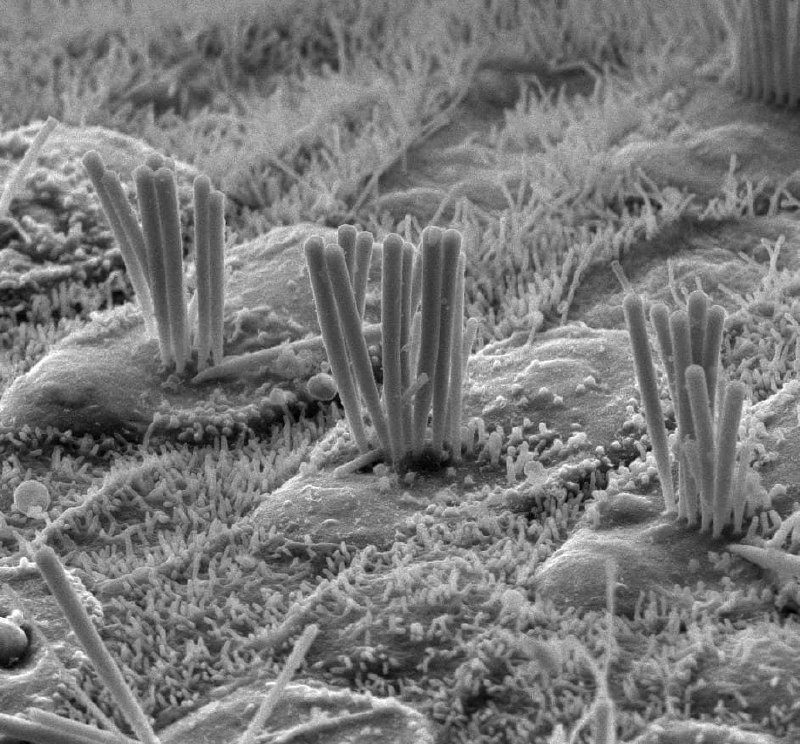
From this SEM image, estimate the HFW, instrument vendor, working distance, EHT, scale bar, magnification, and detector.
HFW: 16.7 µm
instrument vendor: FEI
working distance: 6.2 mm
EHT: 5 kV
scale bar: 5000 nm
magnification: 9 K X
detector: ETD